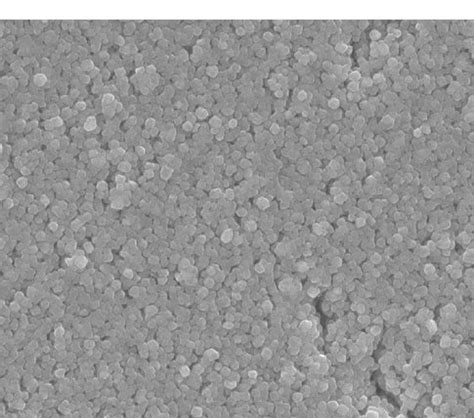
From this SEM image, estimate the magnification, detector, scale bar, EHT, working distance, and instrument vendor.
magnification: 5 K X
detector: SEI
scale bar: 1000 nm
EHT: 15 kV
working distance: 12.2 mm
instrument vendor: JEOL